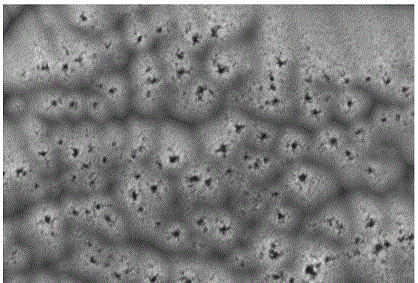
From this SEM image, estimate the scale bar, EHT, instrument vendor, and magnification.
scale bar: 5000 nm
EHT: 10 kV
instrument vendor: JEOL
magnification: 5 K X